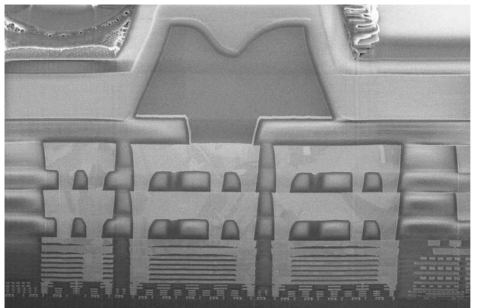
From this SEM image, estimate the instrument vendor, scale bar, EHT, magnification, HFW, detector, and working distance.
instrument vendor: FEI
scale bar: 5000 nm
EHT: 2 kV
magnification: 35 K X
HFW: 11.8 µm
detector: TLD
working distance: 4 mm